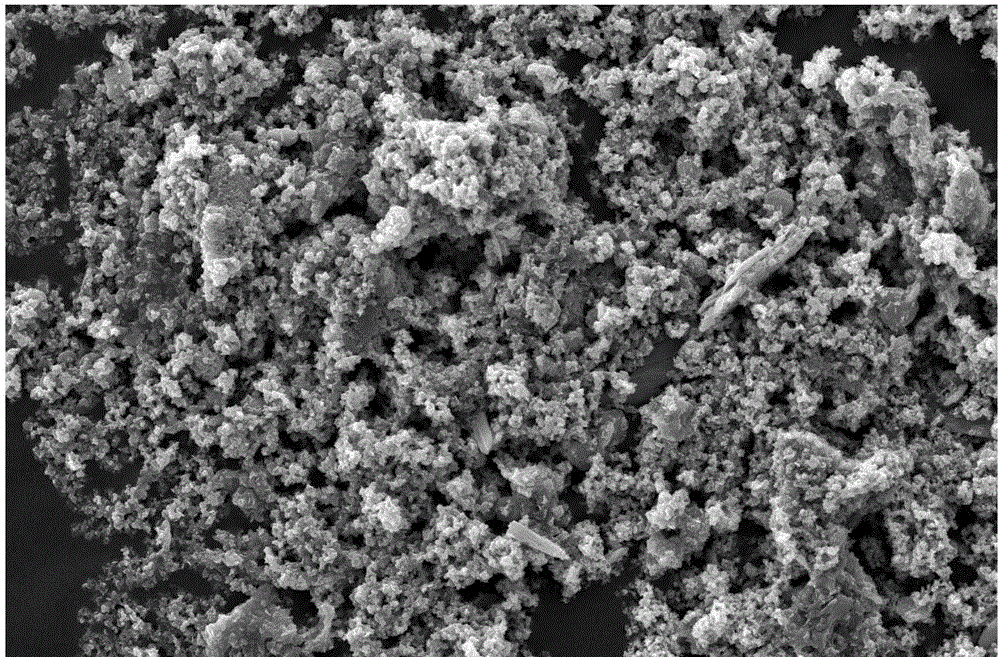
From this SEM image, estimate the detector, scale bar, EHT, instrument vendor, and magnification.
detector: SEI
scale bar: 10000 nm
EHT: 20 kV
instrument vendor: JEOL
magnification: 1 K X